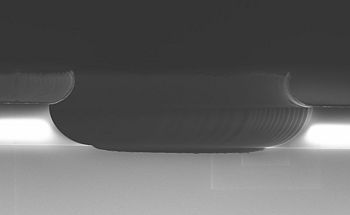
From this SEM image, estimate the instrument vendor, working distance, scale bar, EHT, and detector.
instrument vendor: Zeiss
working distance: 4.9 mm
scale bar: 1000 nm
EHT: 10 kV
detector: InLens